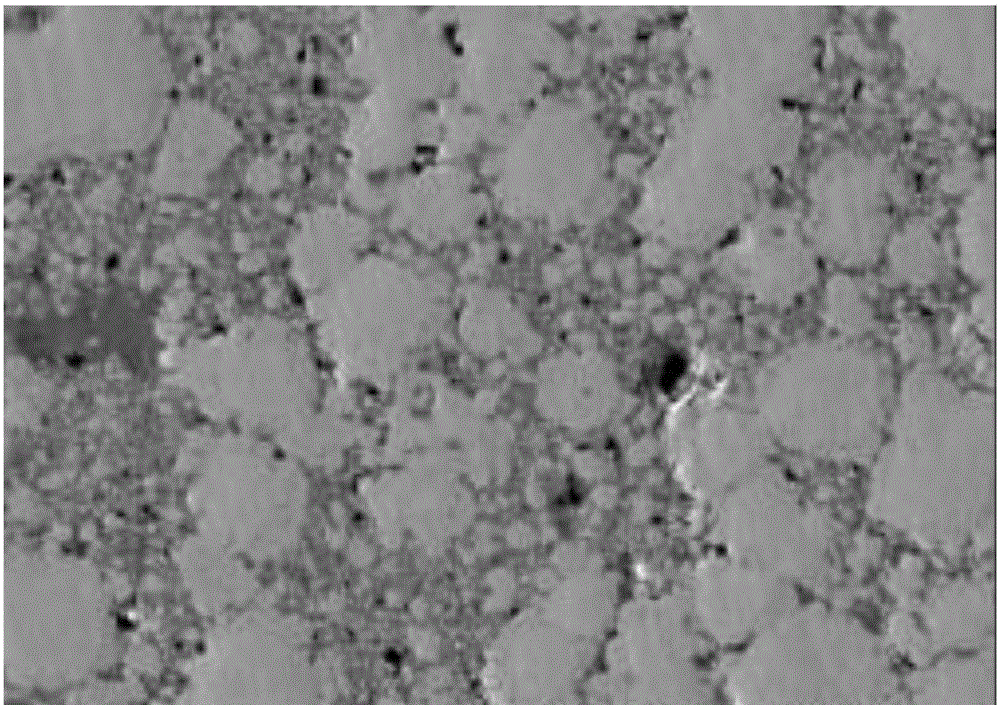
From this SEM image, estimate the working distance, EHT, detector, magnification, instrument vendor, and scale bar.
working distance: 15.1 mm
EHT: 20 kV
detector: LEI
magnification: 2 K X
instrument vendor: JEOL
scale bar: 10000 nm